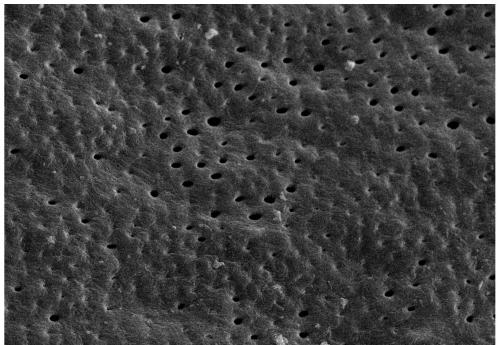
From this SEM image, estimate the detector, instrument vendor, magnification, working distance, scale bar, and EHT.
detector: SE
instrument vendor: Hitachi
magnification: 1 K X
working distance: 9.6 mm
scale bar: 50000 nm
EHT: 20 kV